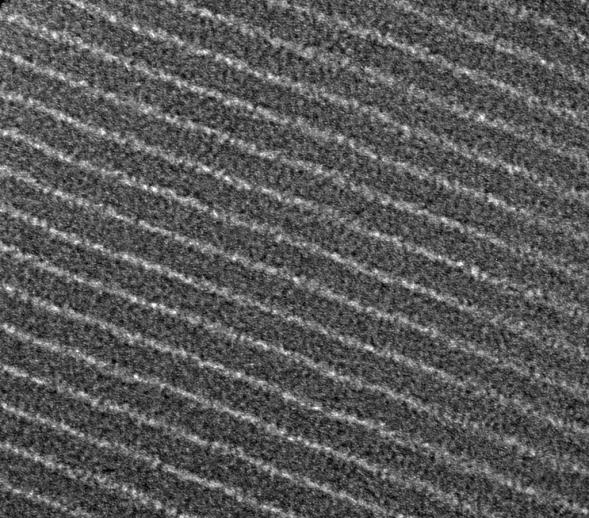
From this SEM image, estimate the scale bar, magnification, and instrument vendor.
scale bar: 10 nm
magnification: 265 K X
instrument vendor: JEOL